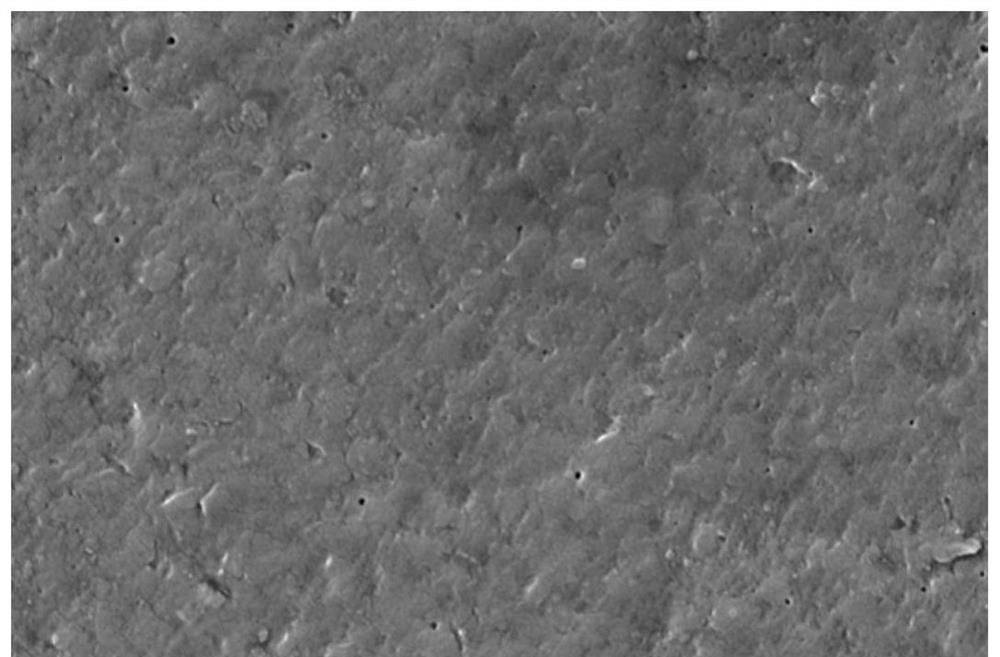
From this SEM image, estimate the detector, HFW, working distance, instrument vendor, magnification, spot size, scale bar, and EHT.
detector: ETD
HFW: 10.4 µm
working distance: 7.9 mm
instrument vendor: FEI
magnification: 40 K X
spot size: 3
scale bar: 2000 nm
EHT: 5 kV